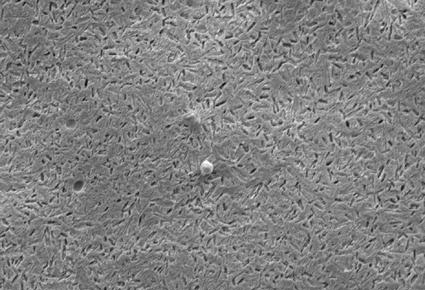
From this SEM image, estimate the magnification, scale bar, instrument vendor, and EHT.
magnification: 3 K X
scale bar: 5000 nm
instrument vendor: JEOL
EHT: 10 kV